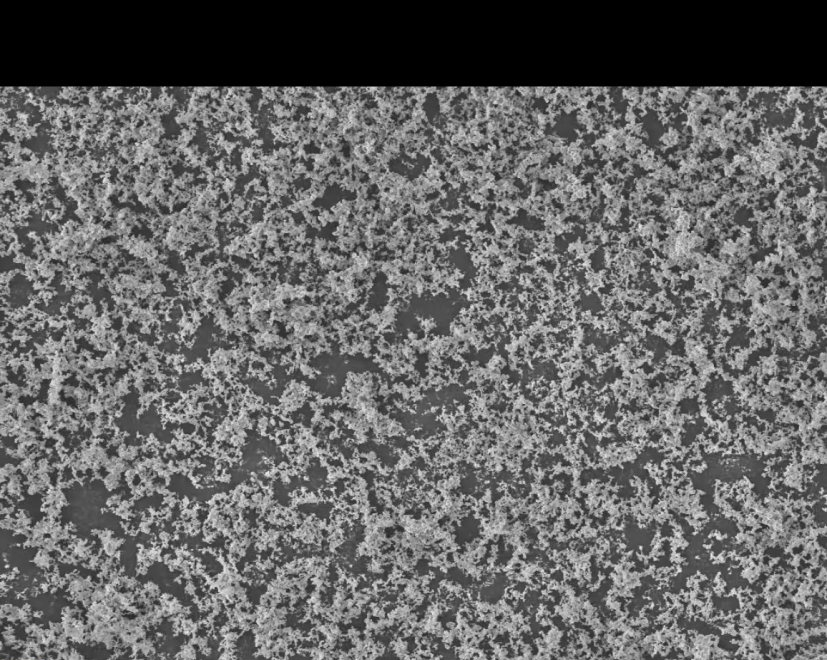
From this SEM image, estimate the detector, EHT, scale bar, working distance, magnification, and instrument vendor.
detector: InLens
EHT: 5 kV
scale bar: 10000 nm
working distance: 4.1 mm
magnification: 1 K X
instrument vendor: Zeiss